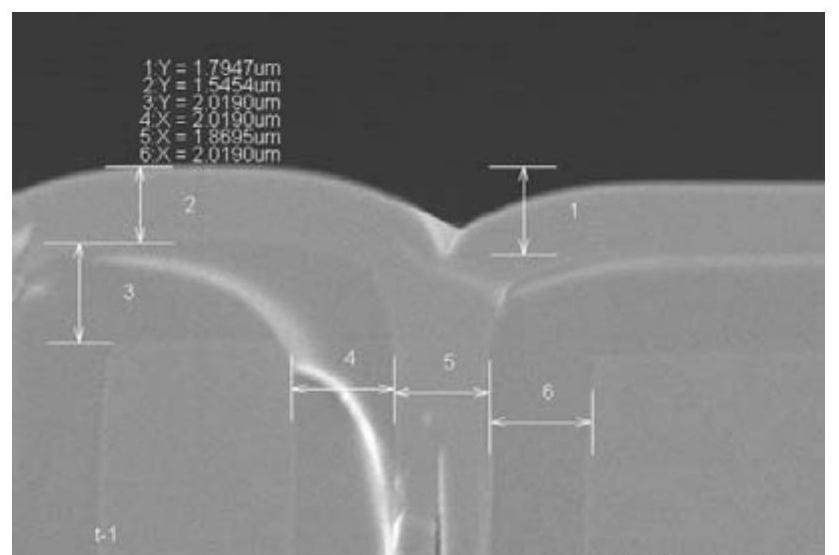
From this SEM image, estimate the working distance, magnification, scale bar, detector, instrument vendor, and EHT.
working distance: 8.2 mm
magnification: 7.96 K X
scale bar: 5000 nm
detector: SE(M)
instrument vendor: Hitachi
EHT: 5 kV